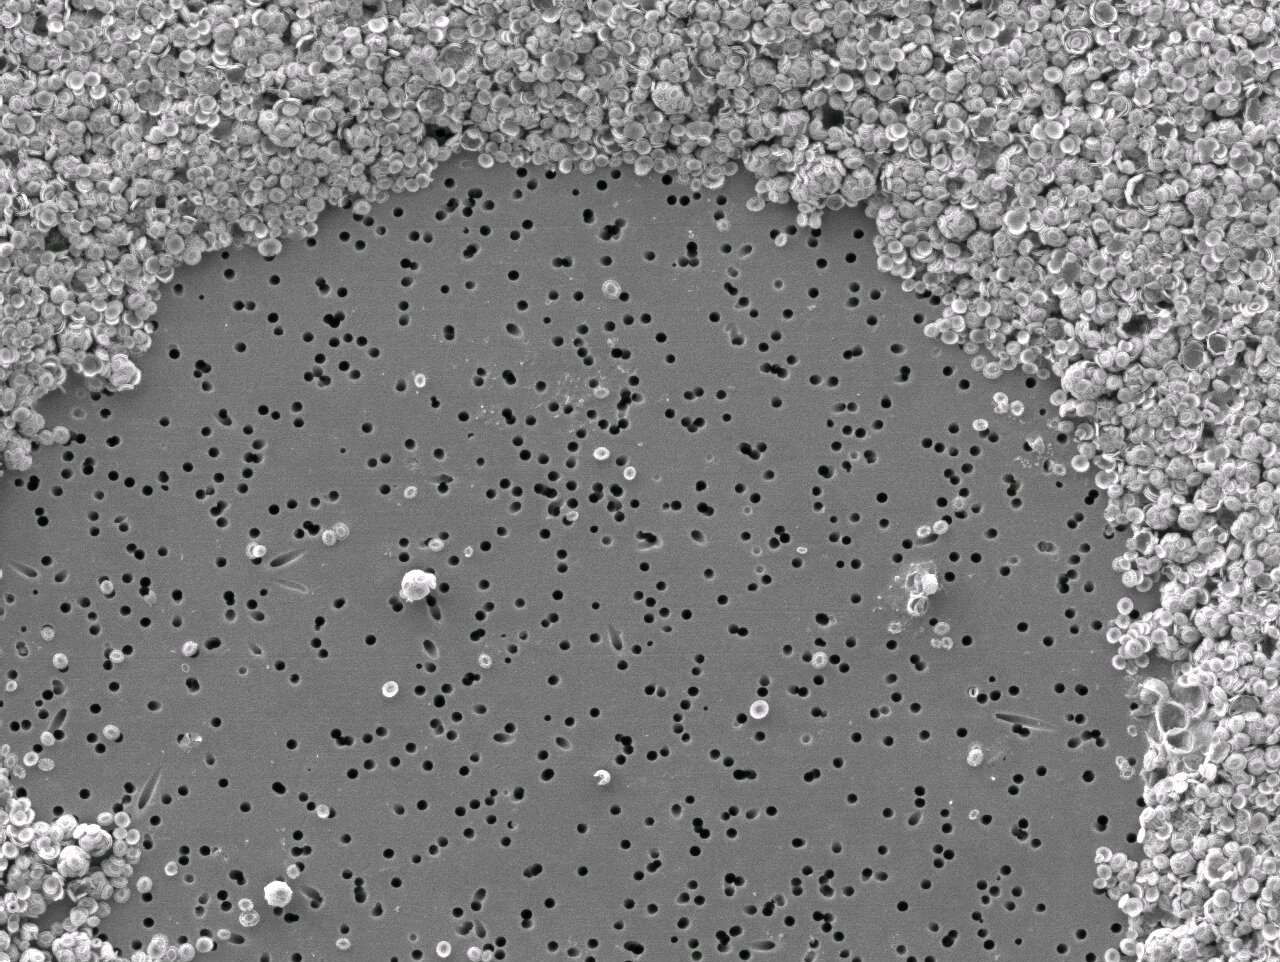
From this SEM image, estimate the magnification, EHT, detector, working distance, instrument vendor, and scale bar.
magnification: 0.5 K X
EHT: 7.5 kV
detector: LEI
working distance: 4.9 mm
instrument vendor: JEOL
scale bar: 10000 nm